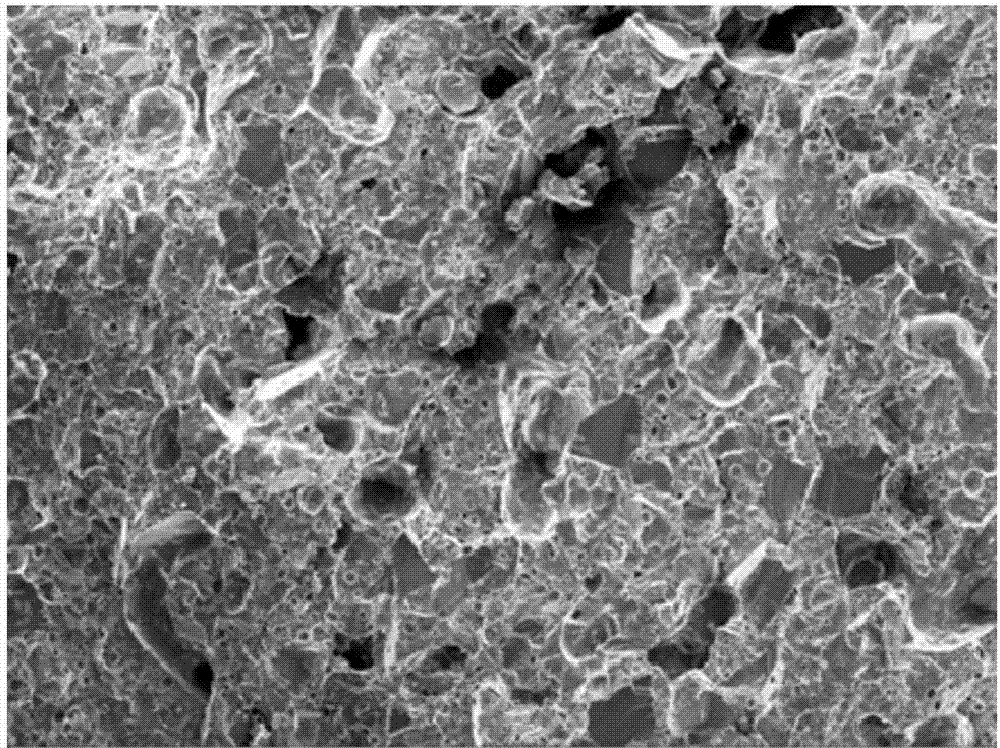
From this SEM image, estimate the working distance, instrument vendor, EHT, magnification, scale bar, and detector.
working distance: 29.4 mm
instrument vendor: Zeiss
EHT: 15 kV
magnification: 0.2 K X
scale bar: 100000 nm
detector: SE2